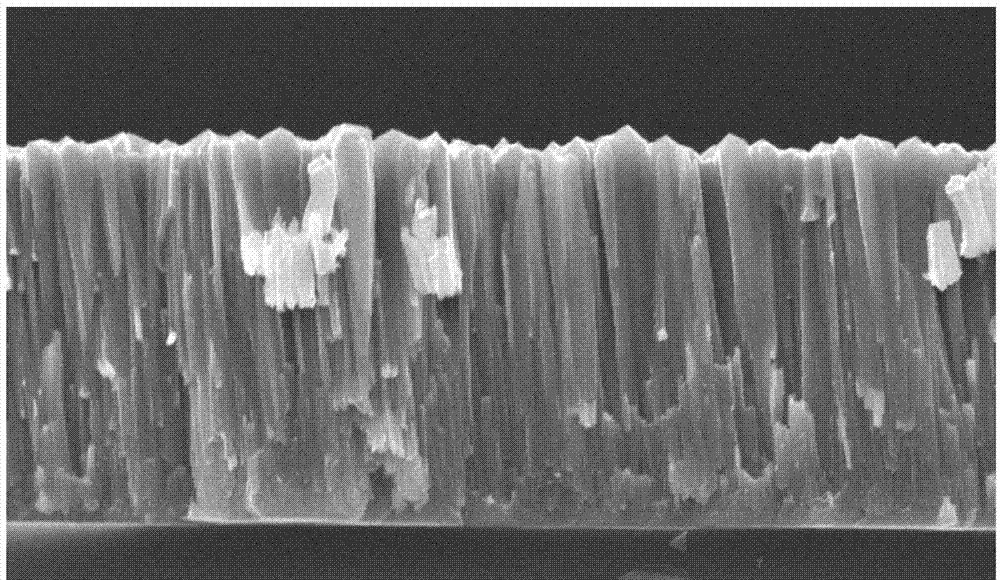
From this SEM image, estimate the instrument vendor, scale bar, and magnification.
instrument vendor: FEI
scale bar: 5000 nm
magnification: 5 K X